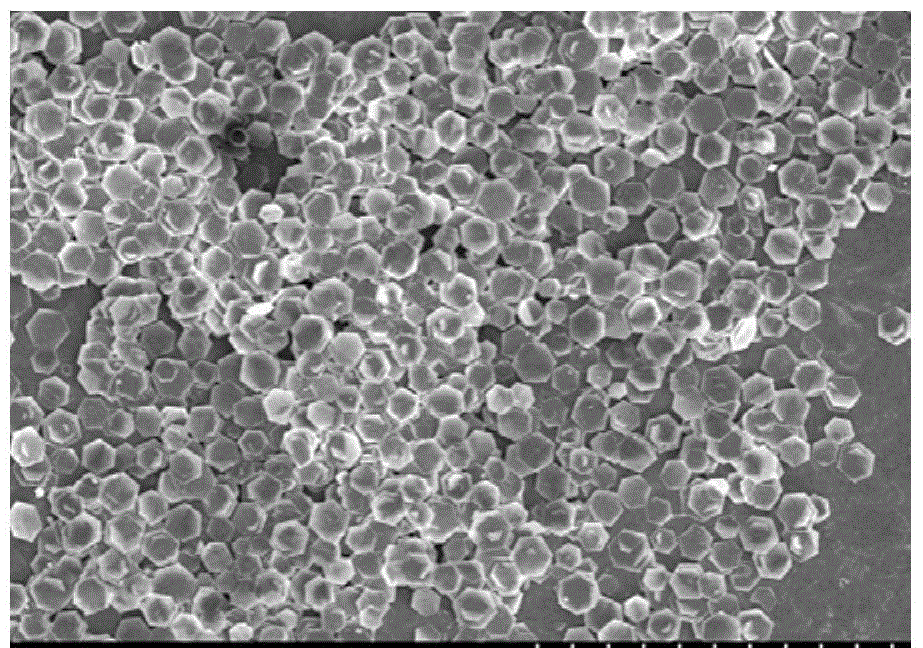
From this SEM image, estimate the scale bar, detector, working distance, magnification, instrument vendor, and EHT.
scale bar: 50000 nm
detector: SE(M)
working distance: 7.3 mm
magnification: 1 K X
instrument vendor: Hitachi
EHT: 5 kV